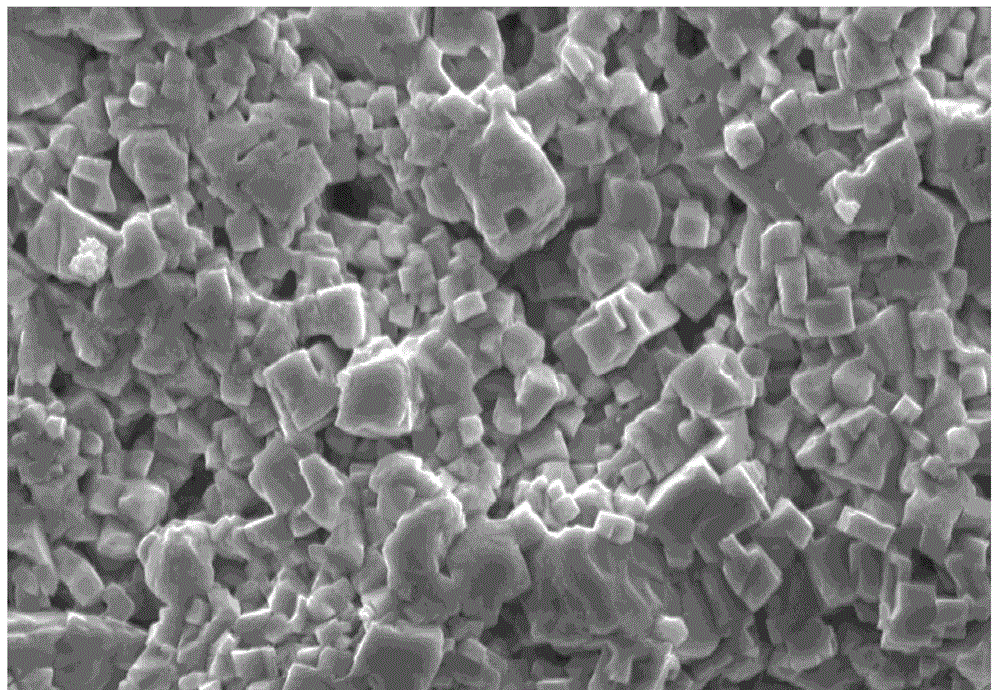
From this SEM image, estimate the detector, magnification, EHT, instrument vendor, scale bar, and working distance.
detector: SE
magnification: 3 K X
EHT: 15 kV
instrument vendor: Hitachi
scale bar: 10000 nm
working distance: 31.5 mm